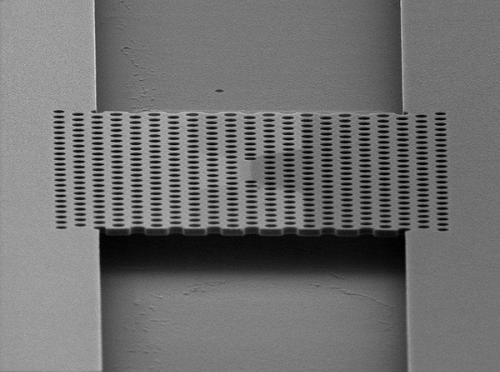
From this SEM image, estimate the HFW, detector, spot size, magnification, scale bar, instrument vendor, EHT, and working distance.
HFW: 11.7 µm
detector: SE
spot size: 3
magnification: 25 K X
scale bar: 2000 nm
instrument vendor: FEI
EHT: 5 kV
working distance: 7.4 mm